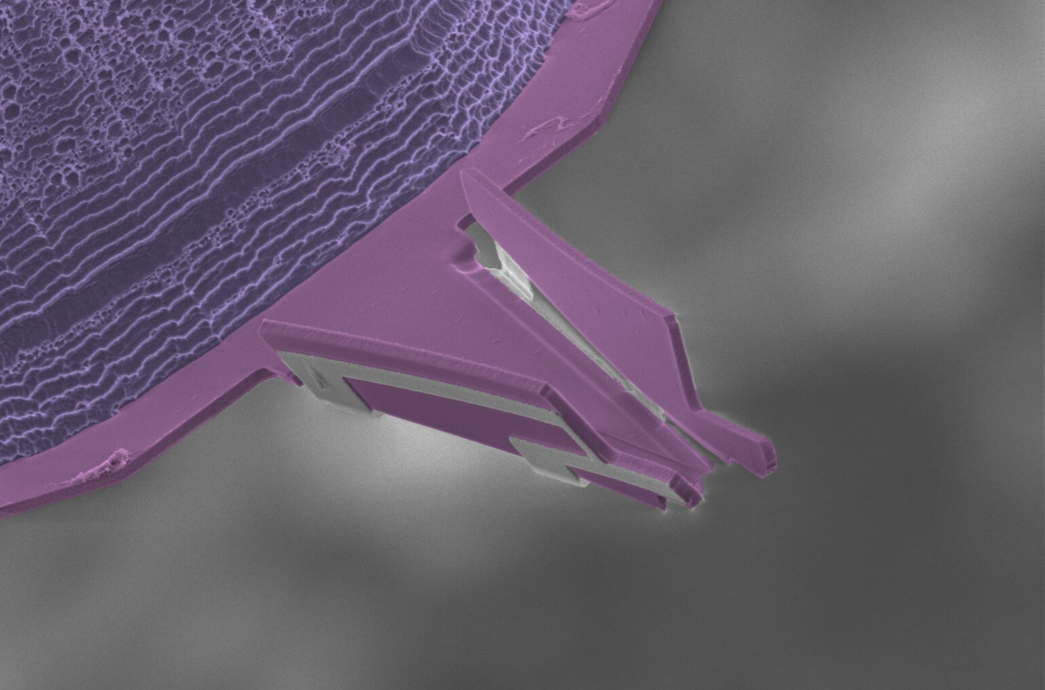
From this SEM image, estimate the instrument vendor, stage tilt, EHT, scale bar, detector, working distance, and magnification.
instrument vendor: FEI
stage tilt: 20°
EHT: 5 kV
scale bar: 30000 nm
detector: ETD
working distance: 36.9 mm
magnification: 1.5 K X